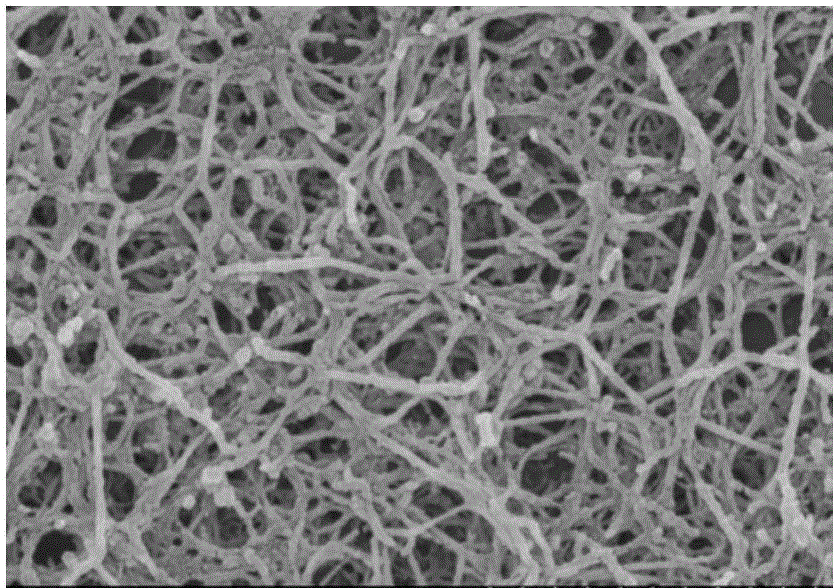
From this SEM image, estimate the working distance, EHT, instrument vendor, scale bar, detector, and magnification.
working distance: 6.9 mm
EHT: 3 kV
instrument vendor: Hitachi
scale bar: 1000 nm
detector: SE(M)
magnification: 50 K X